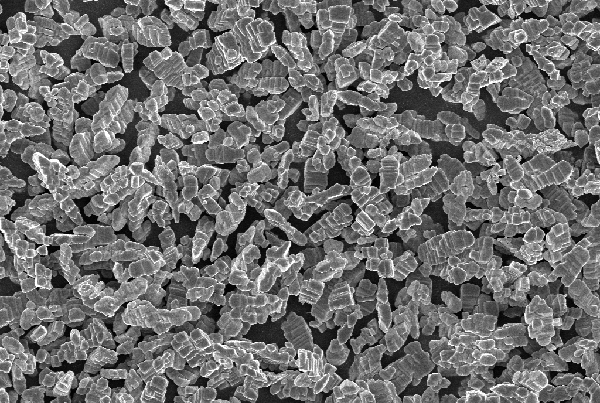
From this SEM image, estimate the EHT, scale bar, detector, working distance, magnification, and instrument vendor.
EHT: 10 kV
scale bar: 10000 nm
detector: InLens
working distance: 5.6 mm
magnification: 1 K X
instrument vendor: Zeiss